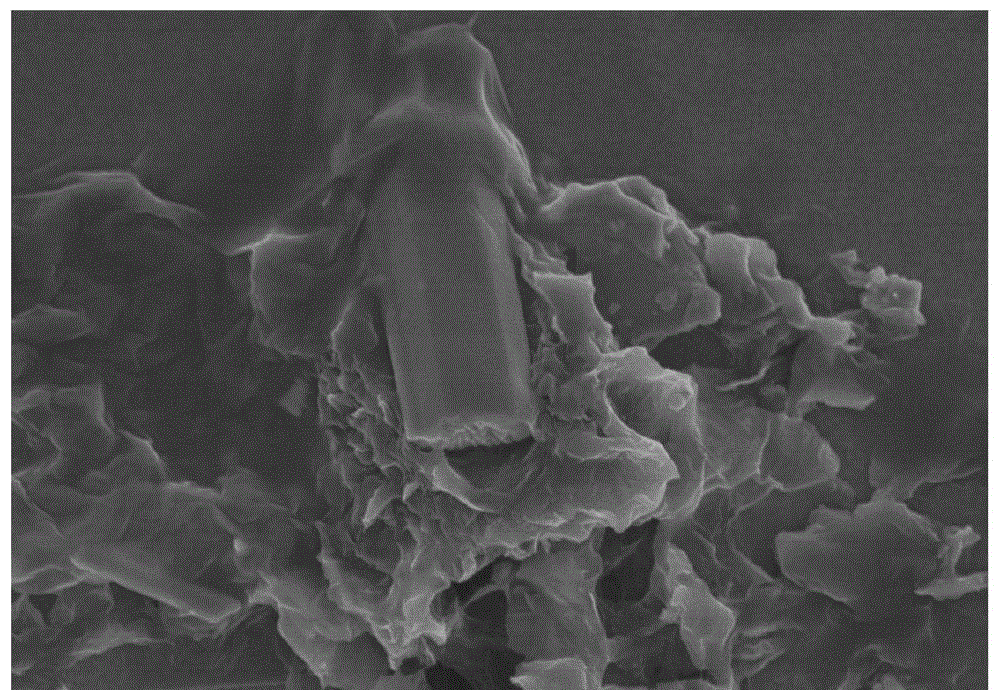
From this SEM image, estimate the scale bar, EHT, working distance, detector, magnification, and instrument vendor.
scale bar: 5000 nm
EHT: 5 kV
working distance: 7.9 mm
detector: SE(UL)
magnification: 10 K X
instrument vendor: Hitachi